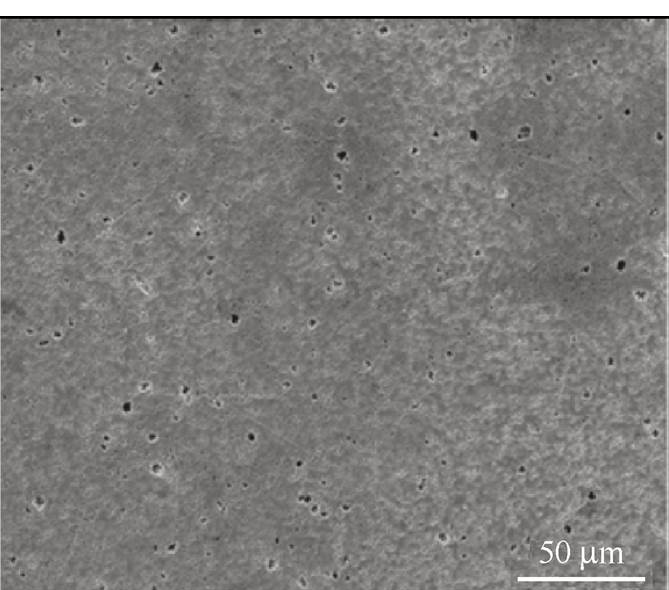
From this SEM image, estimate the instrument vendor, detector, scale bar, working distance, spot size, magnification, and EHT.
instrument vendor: FEI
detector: ETD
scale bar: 50000 nm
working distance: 9.6 mm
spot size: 4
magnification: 1 K X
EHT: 15 kV